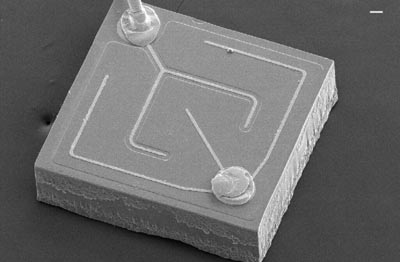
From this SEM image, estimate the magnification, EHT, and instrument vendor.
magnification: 0.13 K X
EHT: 20 kV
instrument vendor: JEOL